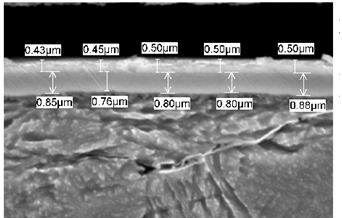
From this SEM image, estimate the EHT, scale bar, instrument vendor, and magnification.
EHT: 20 kV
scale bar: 1000 nm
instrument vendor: JEOL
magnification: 10 K X